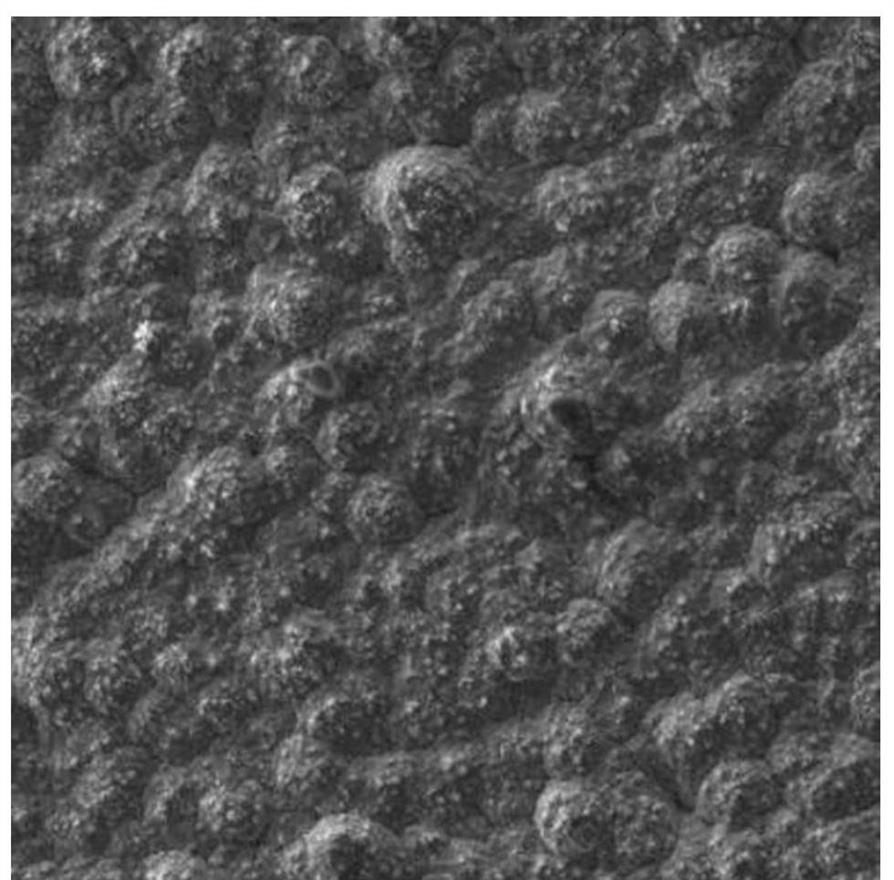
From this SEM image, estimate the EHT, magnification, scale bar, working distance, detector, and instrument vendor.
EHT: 15 kV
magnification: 0.5 K X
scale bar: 50000 nm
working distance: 10.07 mm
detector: SE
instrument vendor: Tescan